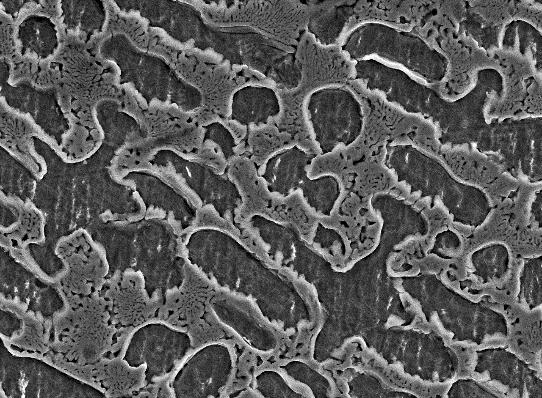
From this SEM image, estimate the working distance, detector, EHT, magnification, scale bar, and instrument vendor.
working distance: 10.1 mm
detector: SEI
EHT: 15 kV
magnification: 2 K X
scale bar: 10000 nm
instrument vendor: JEOL